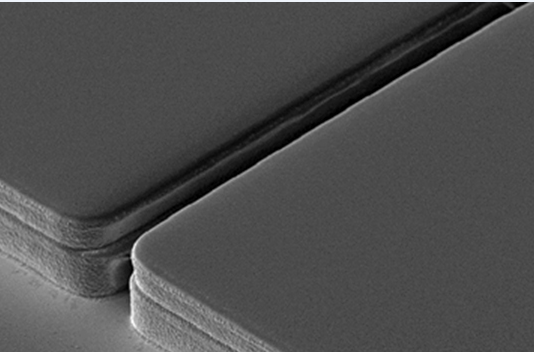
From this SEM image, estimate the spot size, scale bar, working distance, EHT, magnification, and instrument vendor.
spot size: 3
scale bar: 20000 nm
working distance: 17 mm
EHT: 5 kV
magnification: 1 K X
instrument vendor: FEI